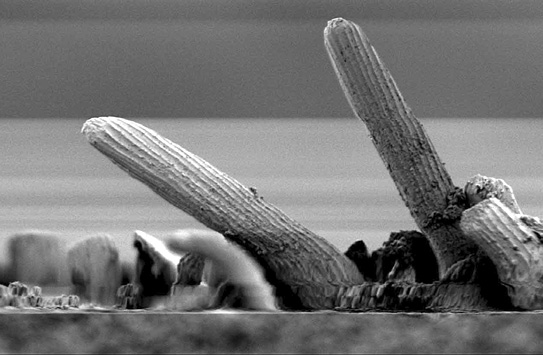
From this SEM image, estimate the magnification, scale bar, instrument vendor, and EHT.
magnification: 0.8 K X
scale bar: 20000 nm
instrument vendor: JEOL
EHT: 1 kV